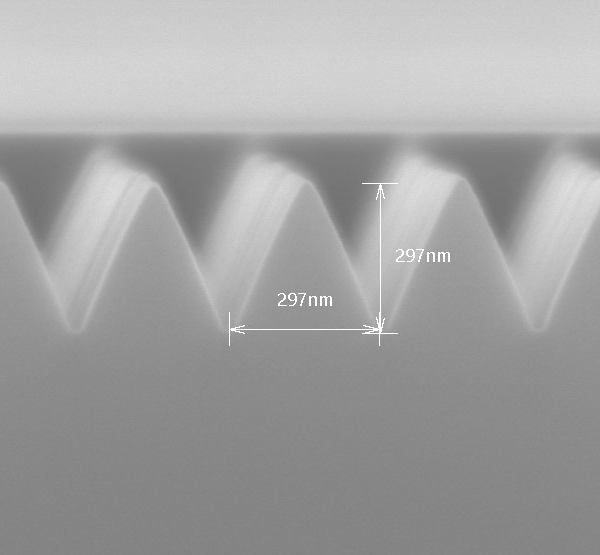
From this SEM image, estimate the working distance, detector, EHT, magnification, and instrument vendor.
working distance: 10.1 mm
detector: SE(U)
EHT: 10 kV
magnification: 80.1 K X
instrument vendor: Hitachi